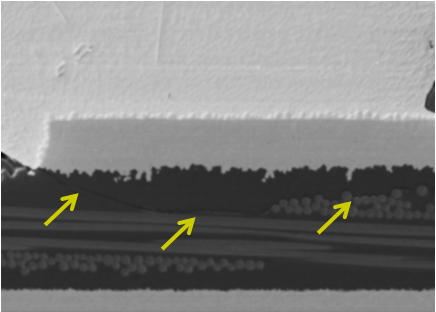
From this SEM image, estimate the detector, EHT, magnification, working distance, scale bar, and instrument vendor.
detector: LEI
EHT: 10 kV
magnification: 0.45 K X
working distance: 15.2 mm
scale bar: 10000 nm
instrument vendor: JEOL